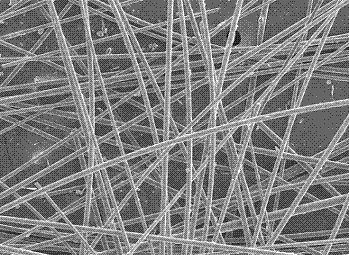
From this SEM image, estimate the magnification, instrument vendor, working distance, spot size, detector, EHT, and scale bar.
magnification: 0.2 K X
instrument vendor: FEI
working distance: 12.4 mm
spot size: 3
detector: SE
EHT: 10 kV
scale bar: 100000 nm